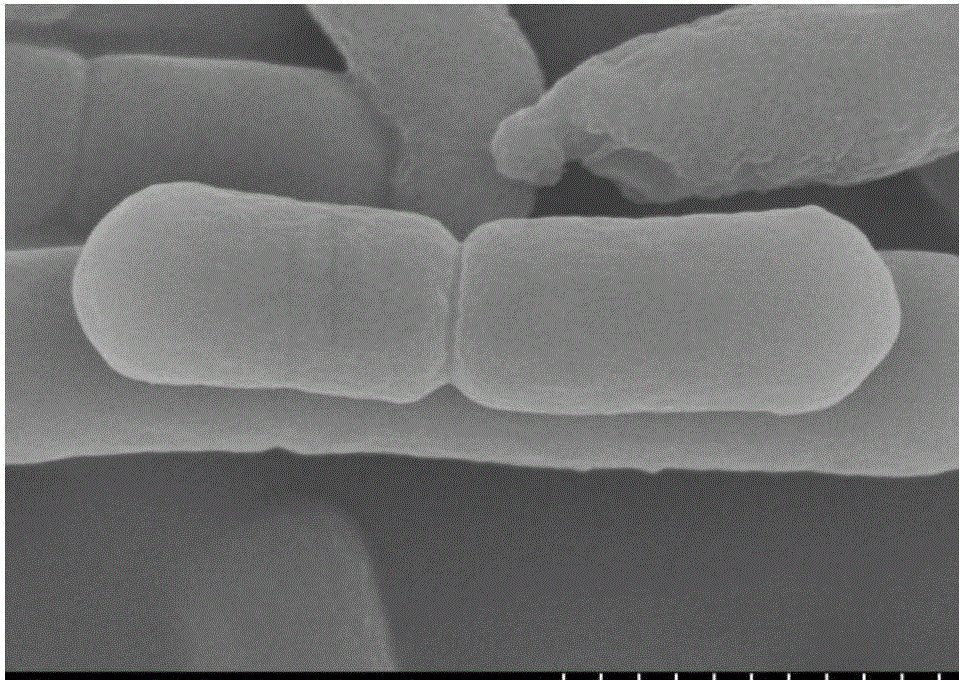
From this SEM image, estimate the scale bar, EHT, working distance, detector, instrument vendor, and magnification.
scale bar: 1000 nm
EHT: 5 kV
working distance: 6.1 mm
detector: SE(M)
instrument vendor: Hitachi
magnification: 50 K X